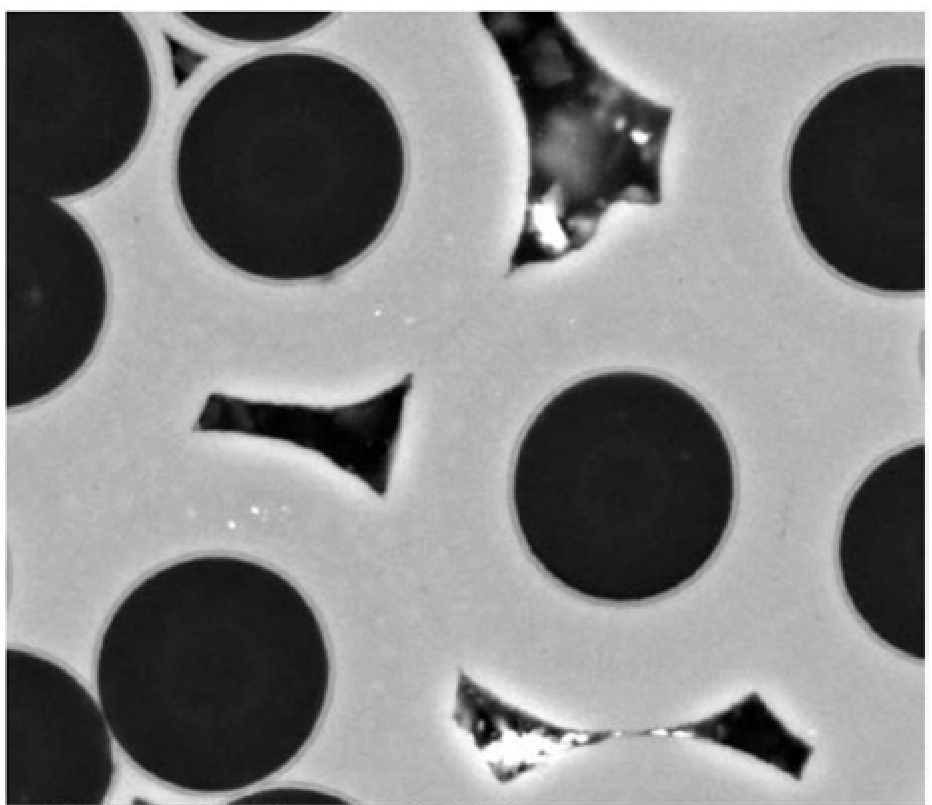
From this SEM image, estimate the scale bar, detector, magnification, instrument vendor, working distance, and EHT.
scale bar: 10000 nm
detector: BSED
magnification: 5 K X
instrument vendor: FEI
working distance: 9.1 mm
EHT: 15 kV